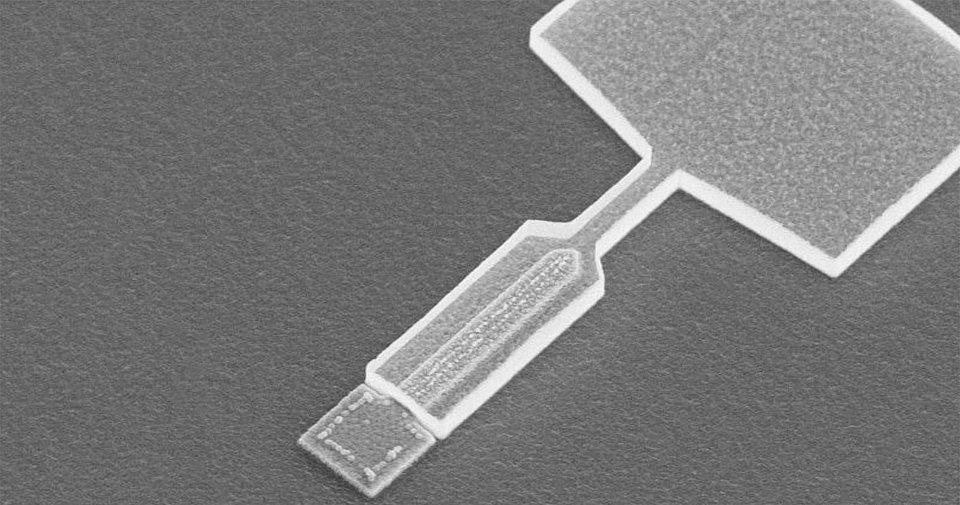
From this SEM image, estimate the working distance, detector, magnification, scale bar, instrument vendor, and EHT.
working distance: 8.2 mm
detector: SE(M)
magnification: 8 K X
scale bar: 3750 nm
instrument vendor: Hitachi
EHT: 10 kV